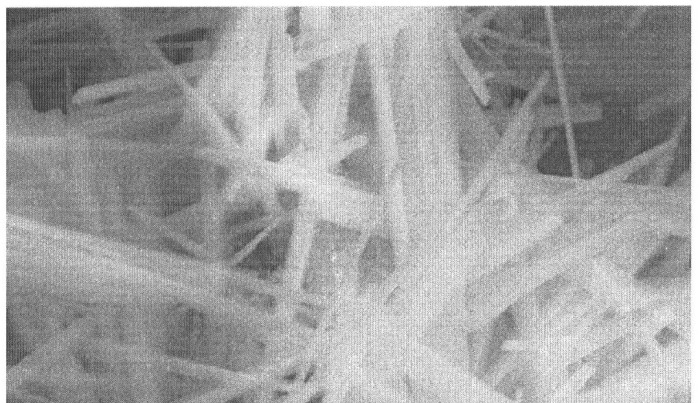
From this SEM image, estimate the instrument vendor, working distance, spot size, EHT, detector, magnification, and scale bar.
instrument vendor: FEI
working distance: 12.3 mm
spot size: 3.8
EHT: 30 kV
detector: GSE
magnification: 5 K X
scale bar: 5000 nm